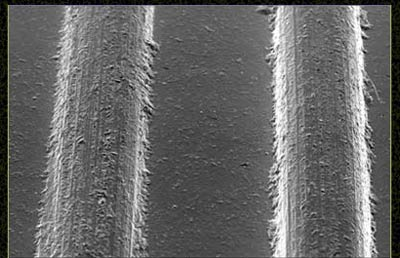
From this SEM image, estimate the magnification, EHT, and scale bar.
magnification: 0.0282 K X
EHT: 10 kV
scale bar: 1000000 nm